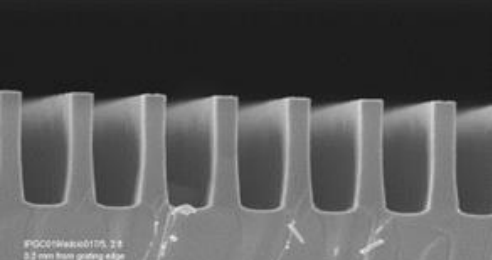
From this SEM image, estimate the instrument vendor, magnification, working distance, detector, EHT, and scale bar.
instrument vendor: Zeiss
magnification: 50 K X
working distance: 2 mm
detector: InLens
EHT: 5 kV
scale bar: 1000 nm